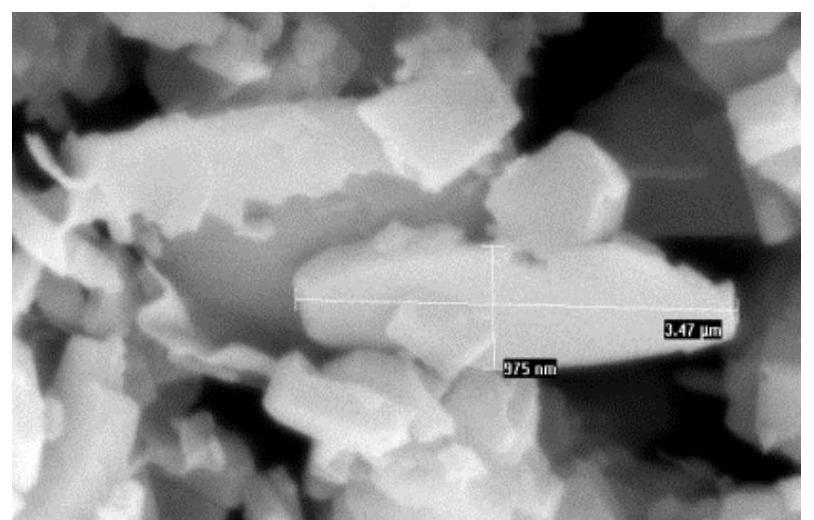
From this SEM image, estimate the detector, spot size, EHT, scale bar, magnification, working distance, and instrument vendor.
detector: SE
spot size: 2.5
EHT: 15 kV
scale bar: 1000 nm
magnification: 20 K X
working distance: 5.3 mm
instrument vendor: FEI